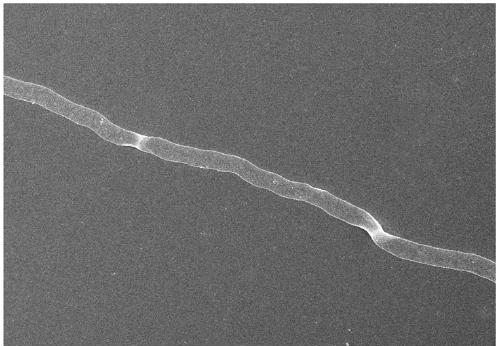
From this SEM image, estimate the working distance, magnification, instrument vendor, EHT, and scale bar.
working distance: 8.2 mm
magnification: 2.2 K X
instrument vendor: Hitachi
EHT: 10 kV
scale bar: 20000 nm